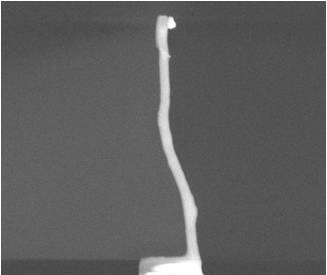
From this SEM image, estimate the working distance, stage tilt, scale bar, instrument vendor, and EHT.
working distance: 2.8 mm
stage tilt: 0°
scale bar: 5000 nm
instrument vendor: FEI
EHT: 10 kV